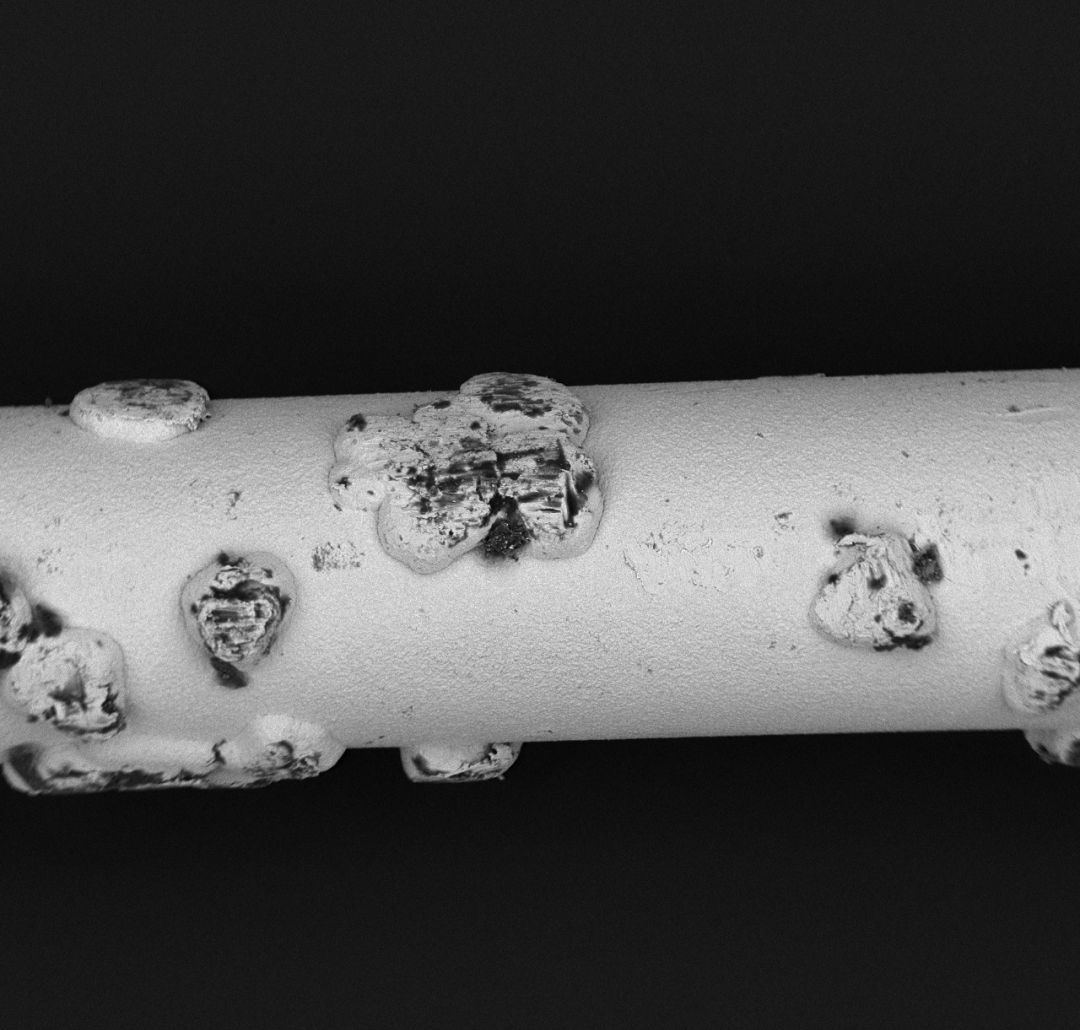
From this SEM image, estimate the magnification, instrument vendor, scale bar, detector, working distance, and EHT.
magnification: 0.5 K X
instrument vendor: other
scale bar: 100000 nm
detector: BSE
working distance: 7.1 mm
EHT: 10 kV